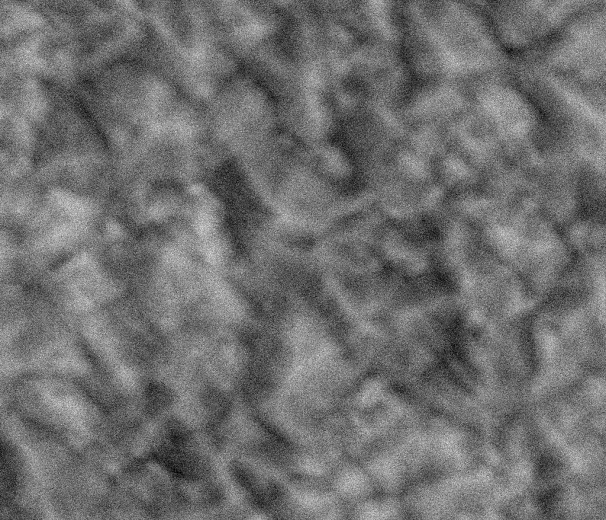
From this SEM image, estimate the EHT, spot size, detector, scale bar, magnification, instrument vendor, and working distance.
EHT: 25 kV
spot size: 5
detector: SSD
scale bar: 10000 nm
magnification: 4 K X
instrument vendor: FEI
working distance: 10.2 mm